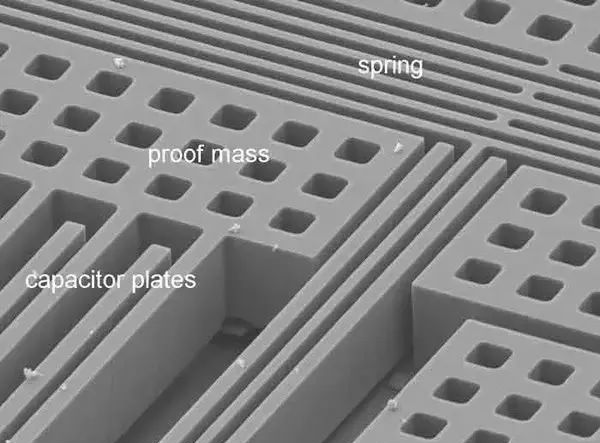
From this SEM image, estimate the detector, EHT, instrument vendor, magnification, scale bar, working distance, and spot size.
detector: SE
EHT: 10 kV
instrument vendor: FEI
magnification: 1.5 K X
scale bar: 20000 nm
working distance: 14.6 mm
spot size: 3